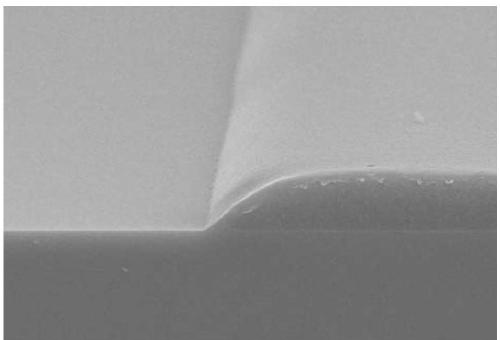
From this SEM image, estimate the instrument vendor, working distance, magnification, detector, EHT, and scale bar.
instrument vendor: Hitachi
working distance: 7.7 mm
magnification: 5 K X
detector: SE(UL)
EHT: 15 kV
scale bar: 10000 nm